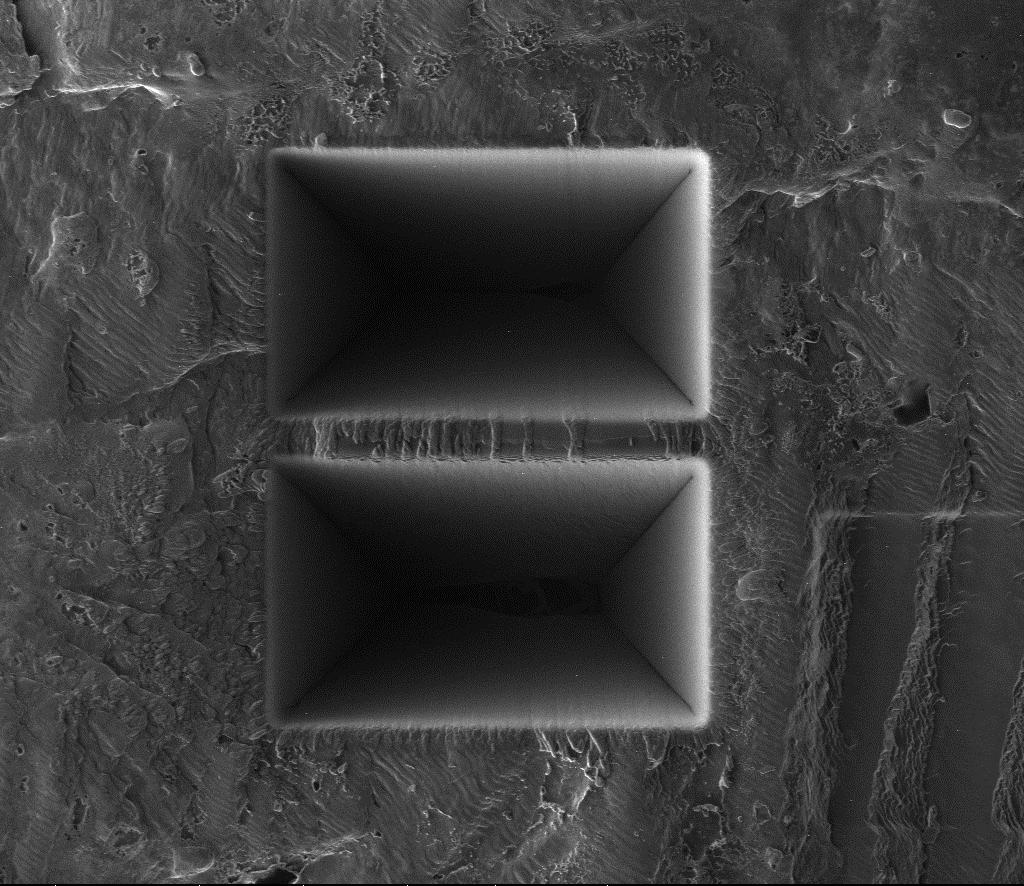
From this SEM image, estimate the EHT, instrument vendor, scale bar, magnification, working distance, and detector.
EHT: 30 kV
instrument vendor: FEI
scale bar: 20000 nm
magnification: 2.5 K X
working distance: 19 mm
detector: CDEM